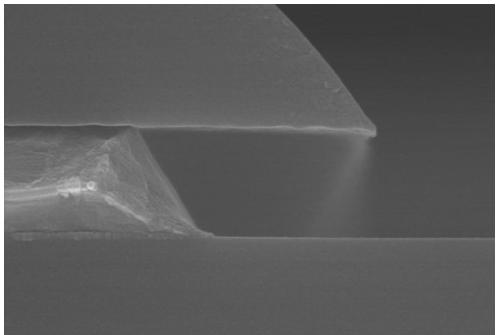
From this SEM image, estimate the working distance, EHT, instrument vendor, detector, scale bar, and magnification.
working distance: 8 mm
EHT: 15 kV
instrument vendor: Hitachi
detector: SE(U,LA0)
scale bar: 500 nm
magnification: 60 K X